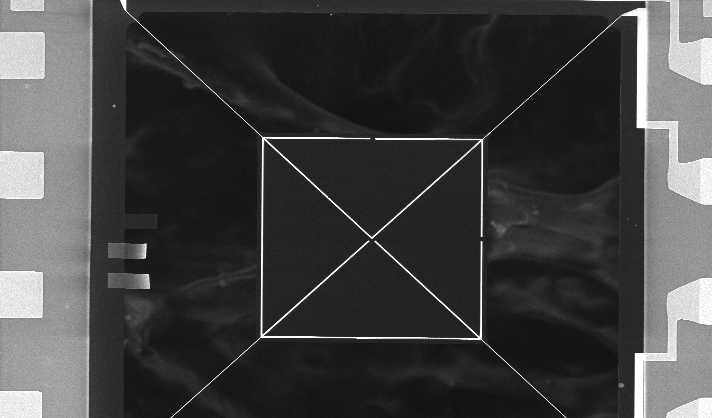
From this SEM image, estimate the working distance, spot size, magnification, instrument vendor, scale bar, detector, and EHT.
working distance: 4.8 mm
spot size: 3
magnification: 0.288 K X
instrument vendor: FEI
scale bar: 100000 nm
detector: TLD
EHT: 15 kV